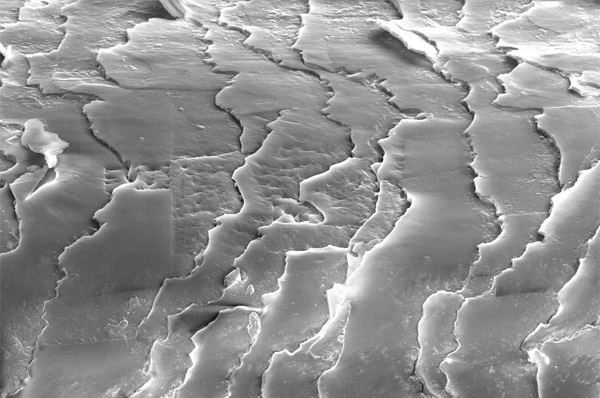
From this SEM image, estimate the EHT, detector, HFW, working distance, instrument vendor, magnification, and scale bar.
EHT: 10 kV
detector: ETD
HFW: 59.2 µm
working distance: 10 mm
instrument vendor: FEI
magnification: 3.5 K X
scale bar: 10000 nm